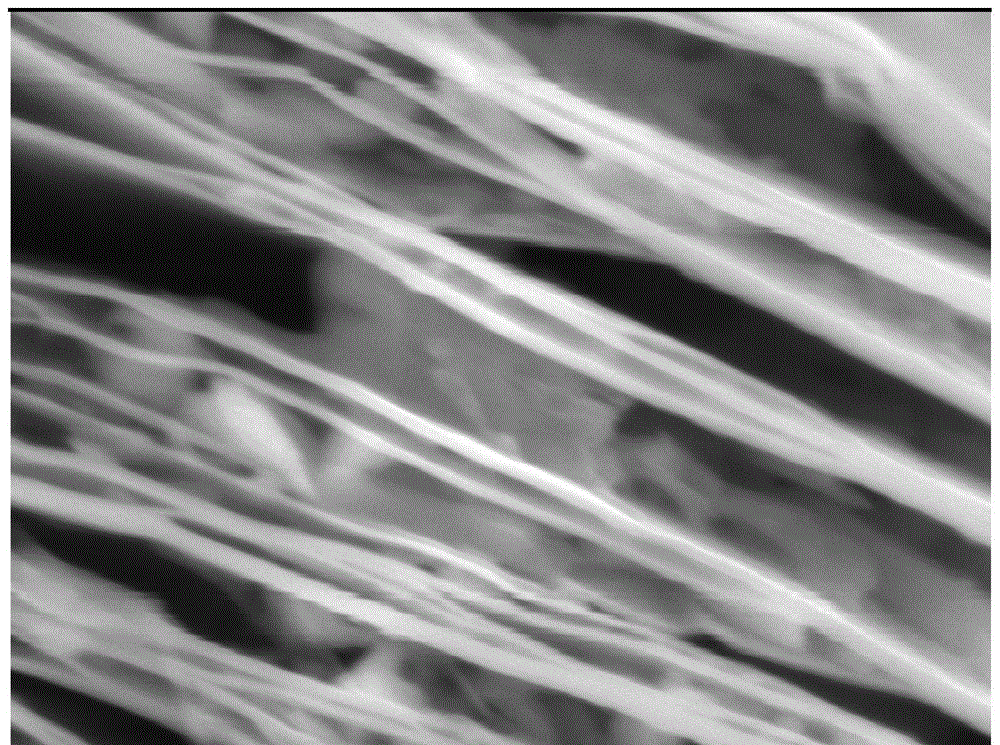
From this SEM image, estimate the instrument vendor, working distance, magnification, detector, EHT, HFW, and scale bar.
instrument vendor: Tescan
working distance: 7.75 mm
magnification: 100 K X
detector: SE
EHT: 10 kV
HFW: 2.77 µm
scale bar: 500 nm